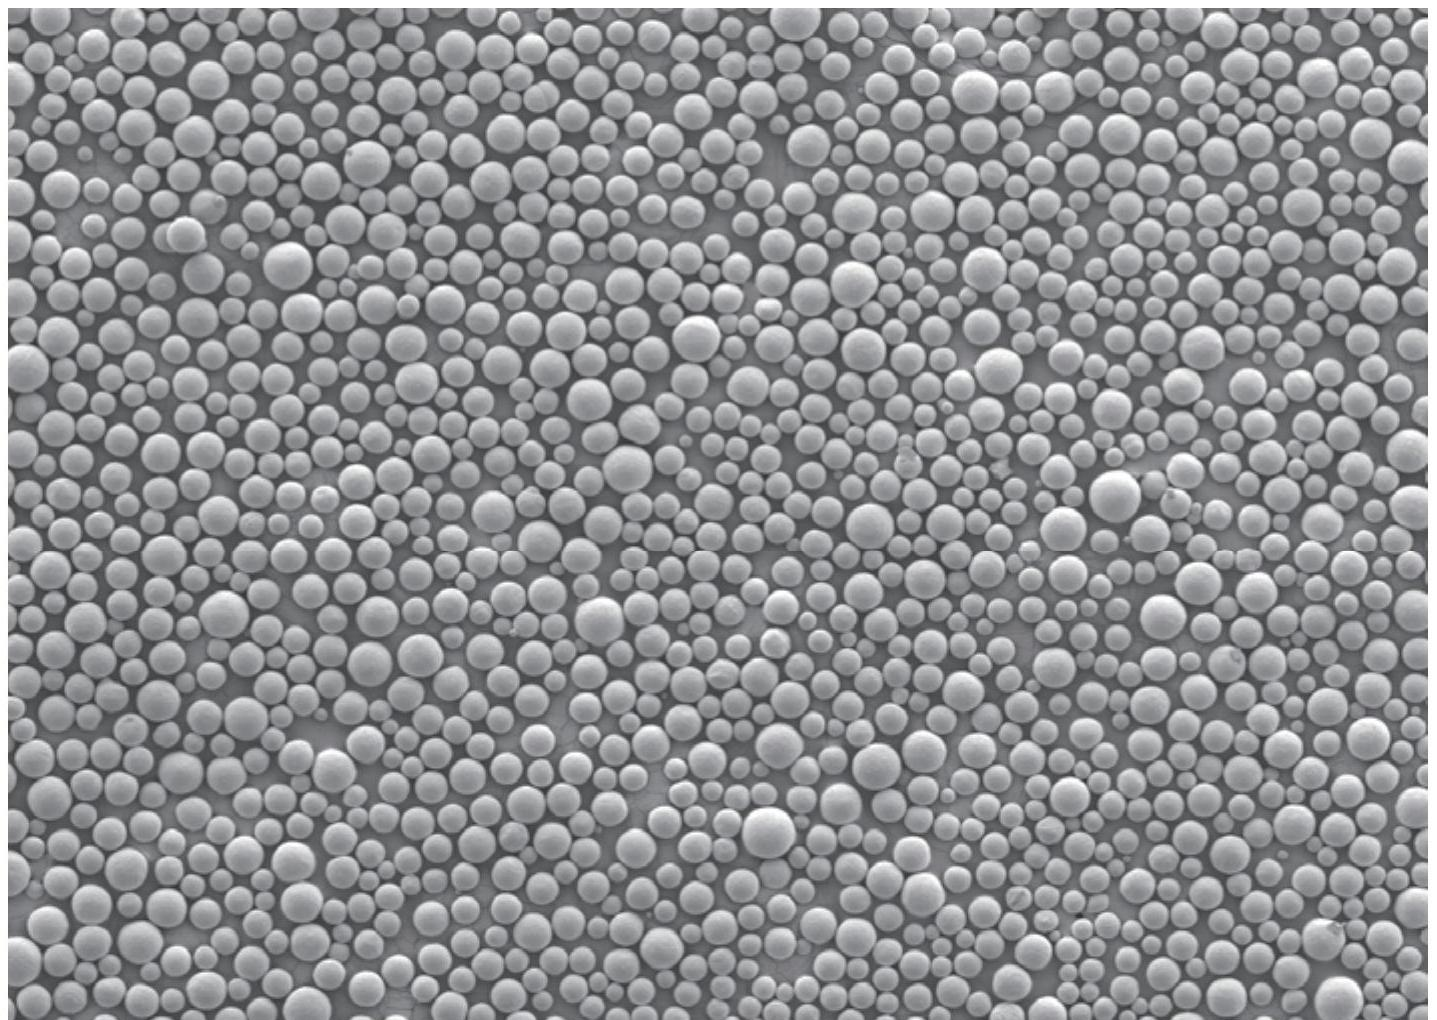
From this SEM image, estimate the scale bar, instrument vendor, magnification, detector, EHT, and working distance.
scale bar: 100000 nm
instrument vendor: JEOL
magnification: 0.05 K X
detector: SEI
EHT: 5 kV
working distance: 8 mm